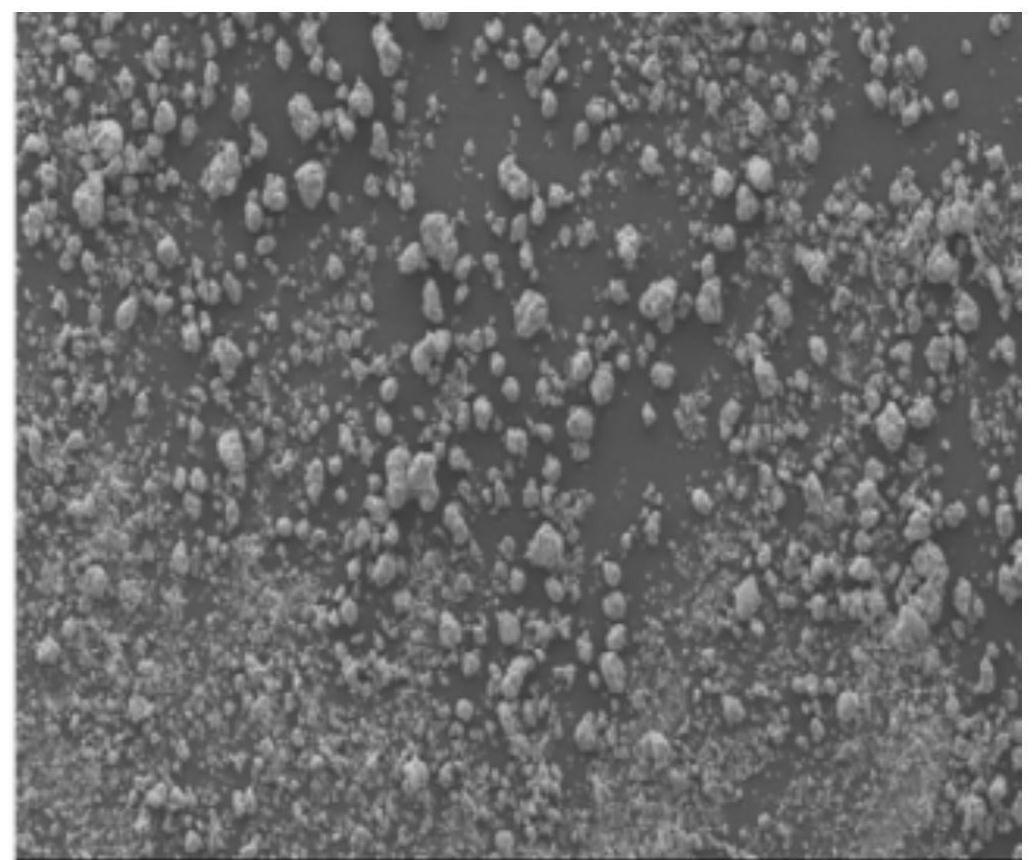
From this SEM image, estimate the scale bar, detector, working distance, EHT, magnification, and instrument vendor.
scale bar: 500000 nm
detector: SE(M)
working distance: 10.2 mm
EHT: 5 kV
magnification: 0.08 K X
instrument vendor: Hitachi